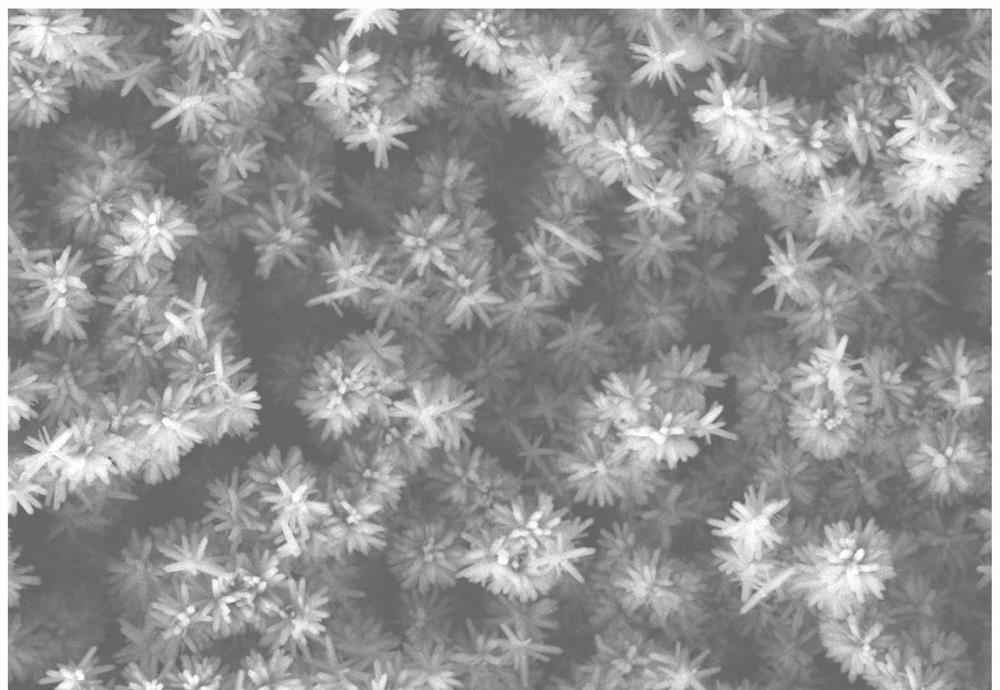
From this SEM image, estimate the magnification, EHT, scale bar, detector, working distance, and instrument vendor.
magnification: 1 K X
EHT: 20 kV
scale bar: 20000 nm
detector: SE2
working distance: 5.7 mm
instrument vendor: Zeiss